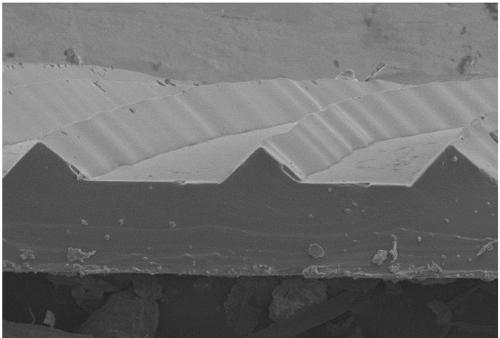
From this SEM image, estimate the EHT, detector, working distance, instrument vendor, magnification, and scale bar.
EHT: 5 kV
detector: LM(UL)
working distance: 8.4 mm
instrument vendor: Hitachi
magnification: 1 K X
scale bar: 100000 nm